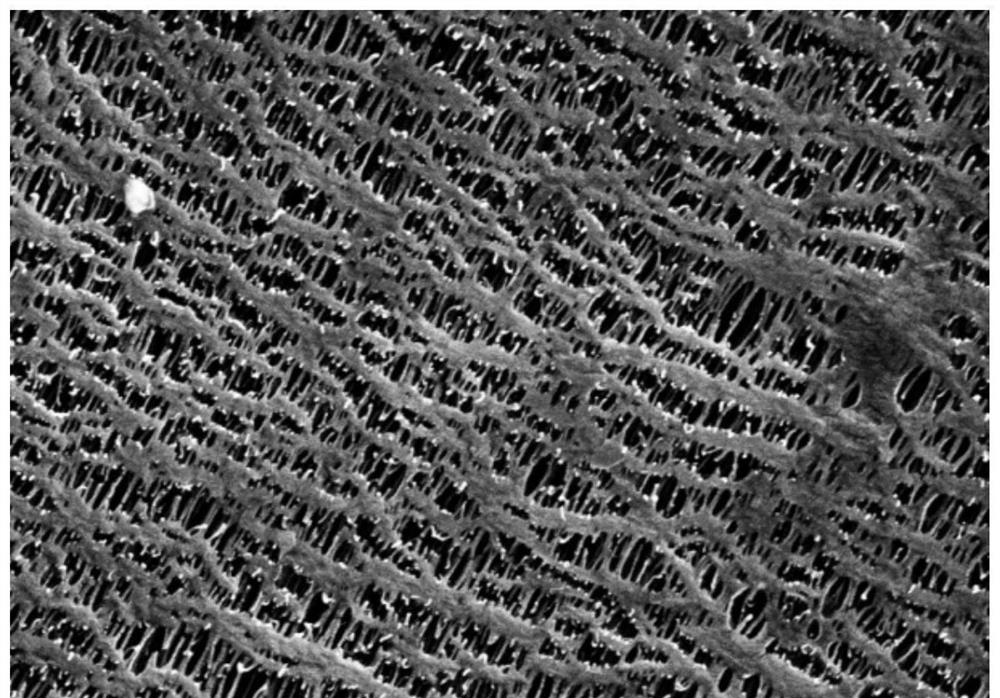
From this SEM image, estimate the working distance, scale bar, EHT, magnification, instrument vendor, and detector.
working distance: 10.2 mm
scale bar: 200 nm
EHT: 5 kV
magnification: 20 K X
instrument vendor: Zeiss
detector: SE2